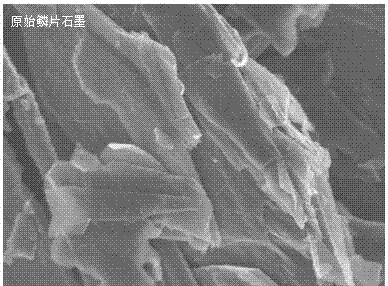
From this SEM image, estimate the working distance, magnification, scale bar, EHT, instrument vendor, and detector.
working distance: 10.8 mm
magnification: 30 K X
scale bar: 1000 nm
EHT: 3 kV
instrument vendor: Hitachi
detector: SE(U)